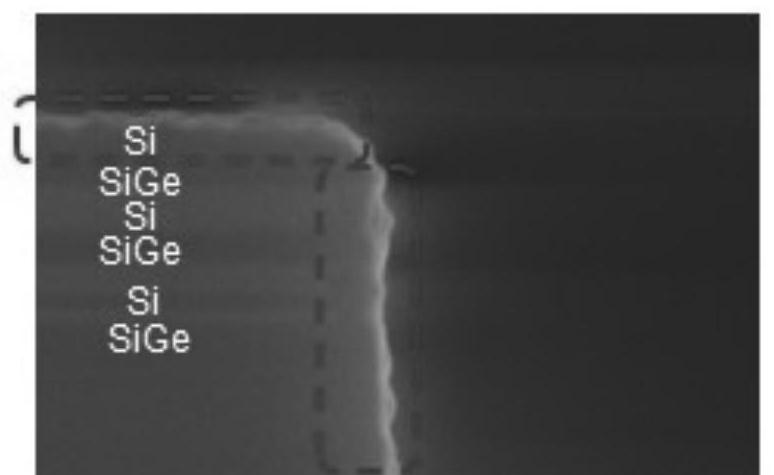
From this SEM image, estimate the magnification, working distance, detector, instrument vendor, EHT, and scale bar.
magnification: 250 K X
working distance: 0.2 mm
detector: SE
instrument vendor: Hitachi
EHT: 3 kV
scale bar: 200 nm